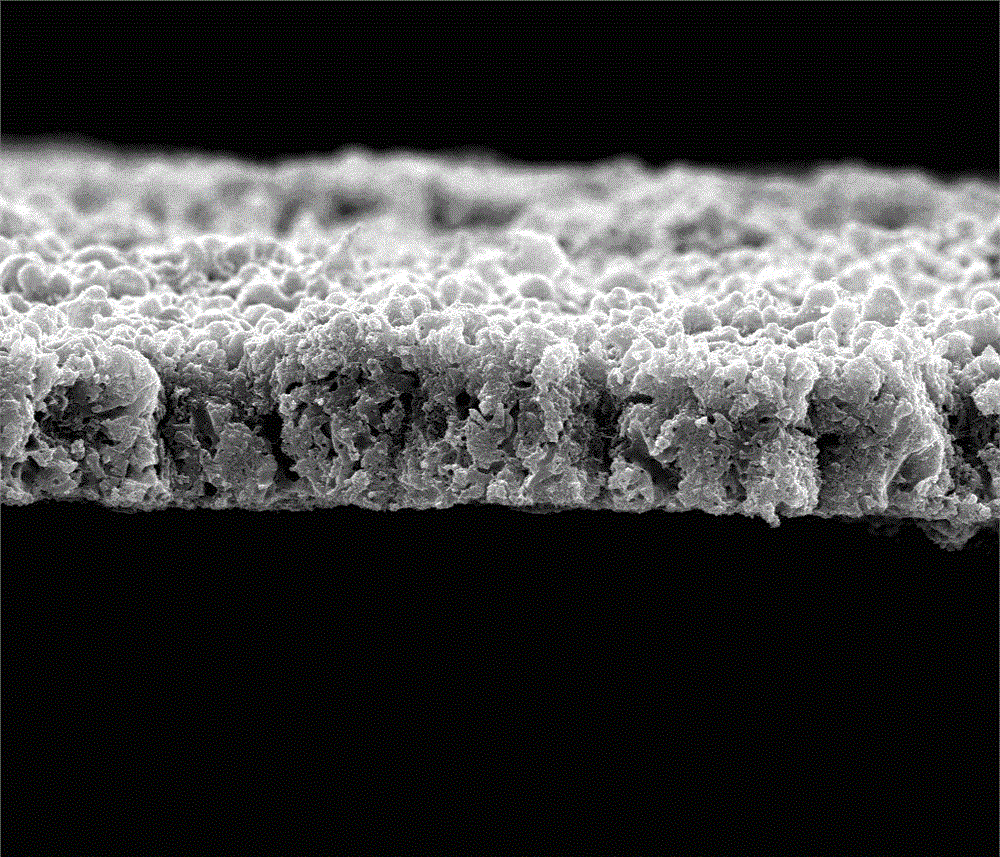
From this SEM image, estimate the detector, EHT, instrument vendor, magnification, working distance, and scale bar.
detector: SE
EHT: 20 kV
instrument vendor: FEI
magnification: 10 K X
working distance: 9.1 mm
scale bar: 10000 nm